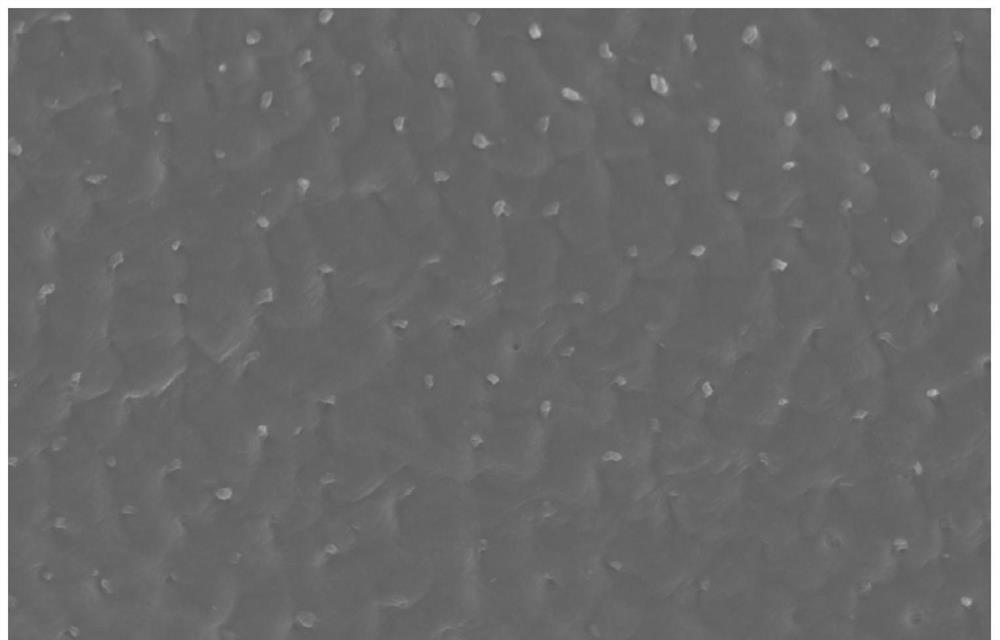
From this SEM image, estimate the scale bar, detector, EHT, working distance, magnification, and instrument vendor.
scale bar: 50000 nm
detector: SE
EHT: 10 kV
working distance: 13.2 mm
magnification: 1 K X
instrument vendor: Hitachi